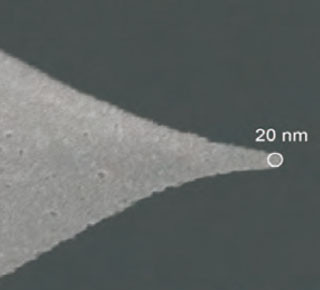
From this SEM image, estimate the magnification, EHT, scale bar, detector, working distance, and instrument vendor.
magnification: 100 K X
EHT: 15 kV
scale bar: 100 nm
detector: SEI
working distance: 6.5 mm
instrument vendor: JEOL